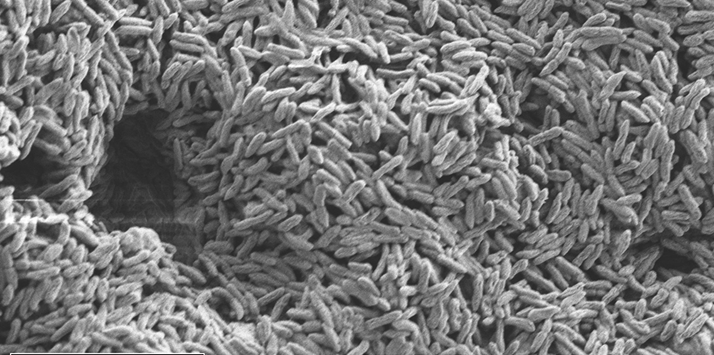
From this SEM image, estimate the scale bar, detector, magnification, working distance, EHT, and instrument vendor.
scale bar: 1000 nm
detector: SE2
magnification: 10 K X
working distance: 4.3 mm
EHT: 2 kV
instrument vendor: Zeiss